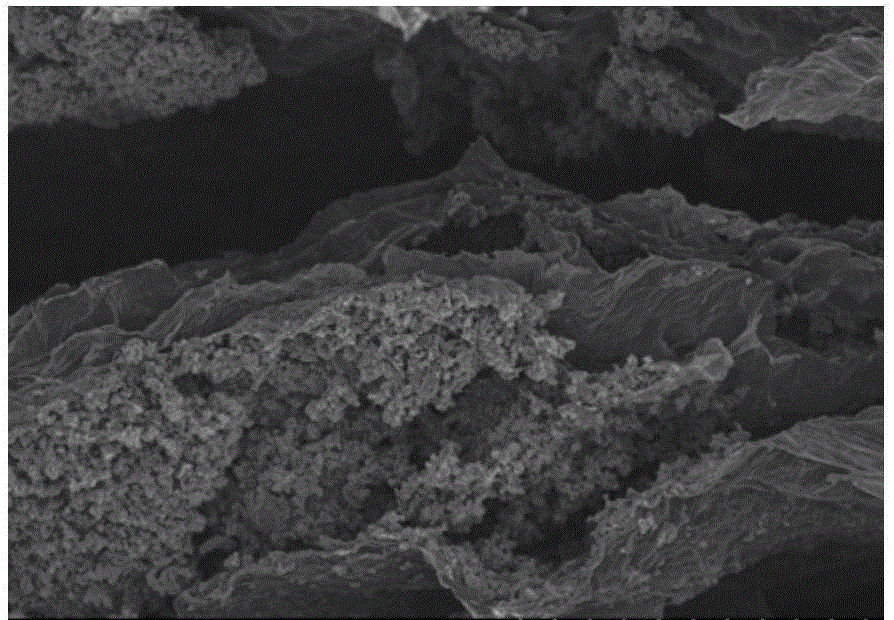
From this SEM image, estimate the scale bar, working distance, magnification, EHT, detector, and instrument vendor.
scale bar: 200000 nm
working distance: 13 mm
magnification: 0.25 K X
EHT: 3 kV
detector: SE(M)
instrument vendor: Hitachi